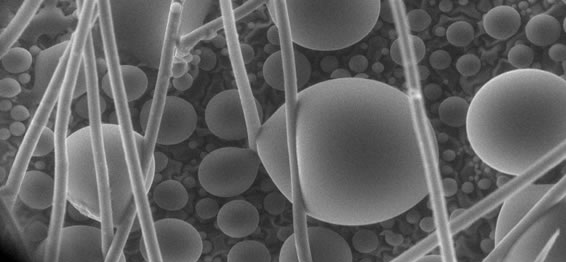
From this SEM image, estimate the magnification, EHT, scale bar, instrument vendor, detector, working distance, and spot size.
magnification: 0.25 K X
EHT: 20 kV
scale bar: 100000 nm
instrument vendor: FEI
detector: GSE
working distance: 8.4 mm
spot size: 5.2